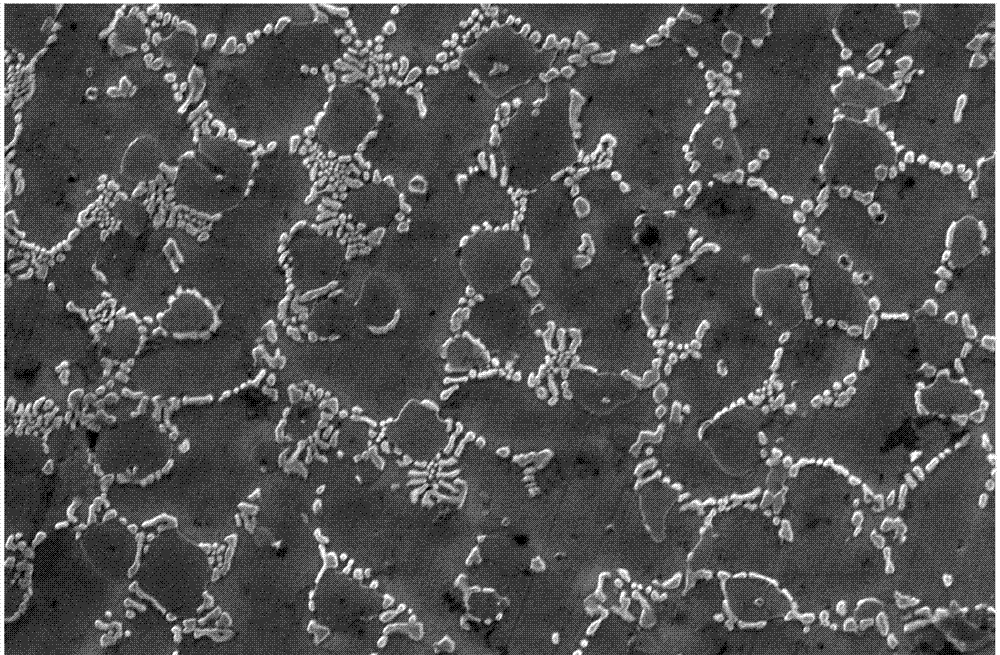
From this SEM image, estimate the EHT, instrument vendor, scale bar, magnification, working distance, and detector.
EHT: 30 kV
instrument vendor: FEI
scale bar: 10000 nm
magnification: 10 K X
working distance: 10 mm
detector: ETD